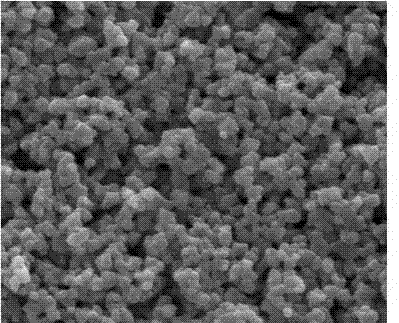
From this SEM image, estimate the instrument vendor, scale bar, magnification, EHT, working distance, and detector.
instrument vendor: FEI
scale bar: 2000 nm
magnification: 50 K X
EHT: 20 kV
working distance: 11.4 mm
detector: SE